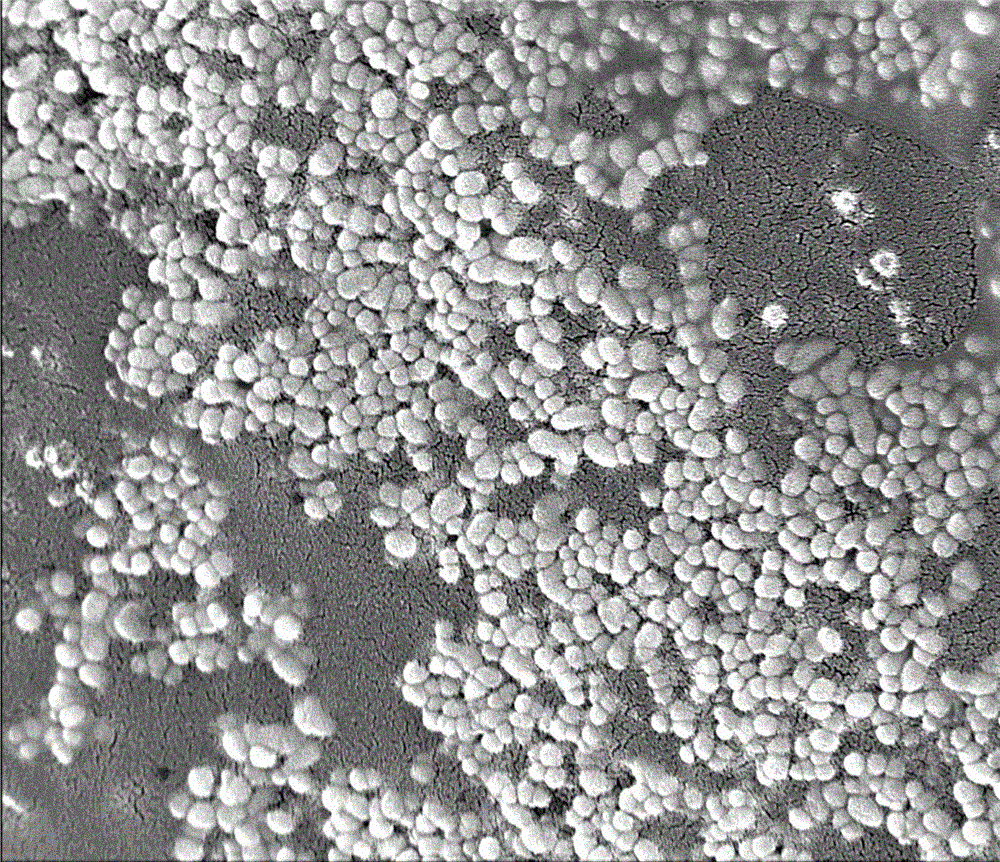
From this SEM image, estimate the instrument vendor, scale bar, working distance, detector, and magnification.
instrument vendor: FEI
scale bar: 2000 nm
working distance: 4.7 mm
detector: TLD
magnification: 30 K X